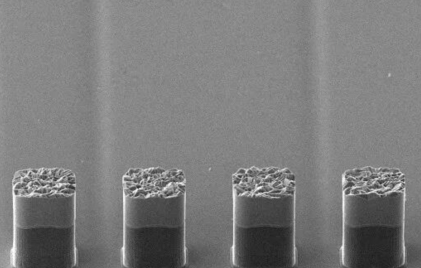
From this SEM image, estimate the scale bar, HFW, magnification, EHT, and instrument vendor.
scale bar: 50000 nm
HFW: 279 µm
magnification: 1 K X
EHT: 20 kV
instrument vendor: Thermo